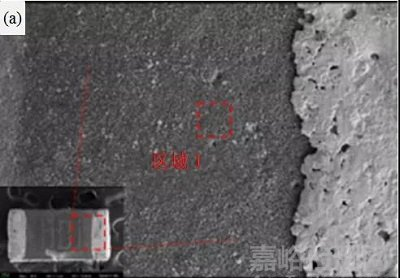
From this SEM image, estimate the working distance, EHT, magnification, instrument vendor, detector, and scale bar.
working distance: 7.1 mm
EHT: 10 kV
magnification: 0.5 K X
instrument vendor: Zeiss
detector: SE2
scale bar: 20000 nm